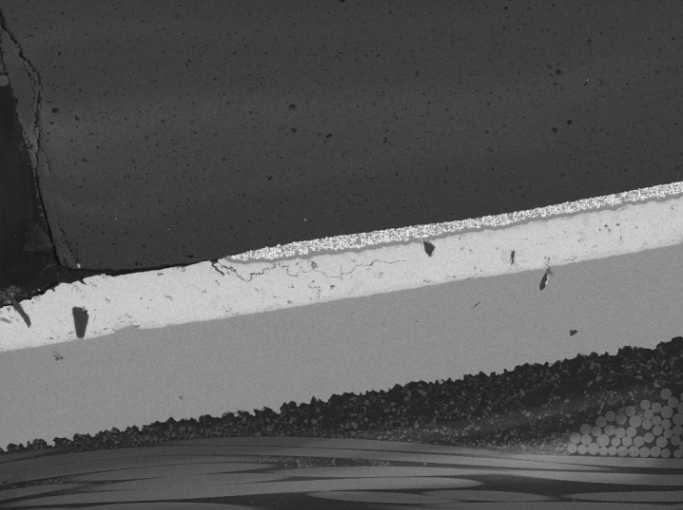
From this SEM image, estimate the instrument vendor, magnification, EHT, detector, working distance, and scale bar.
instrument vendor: Tescan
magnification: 0.504 K X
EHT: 20 kV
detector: BSE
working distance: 14.58 mm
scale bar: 100000 nm